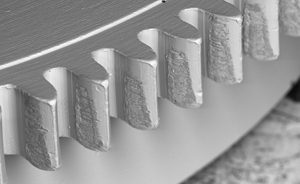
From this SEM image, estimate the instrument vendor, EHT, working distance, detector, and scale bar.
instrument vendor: Tescan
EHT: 20 kV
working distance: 21 mm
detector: BSE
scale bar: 600000 nm